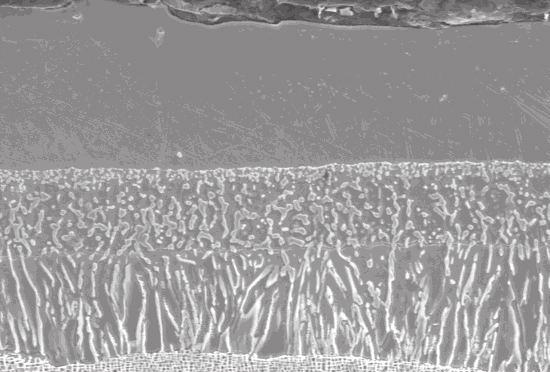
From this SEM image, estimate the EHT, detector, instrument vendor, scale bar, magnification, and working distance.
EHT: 15 kV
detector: SE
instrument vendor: Hitachi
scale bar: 30000 nm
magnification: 1.5 K X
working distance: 11.4 mm